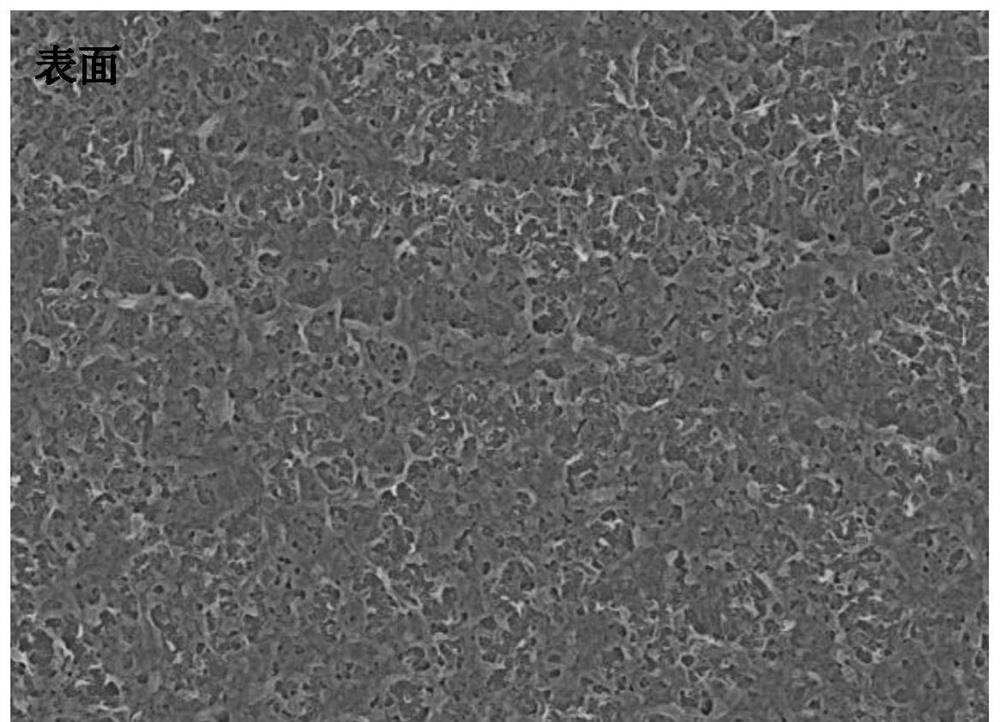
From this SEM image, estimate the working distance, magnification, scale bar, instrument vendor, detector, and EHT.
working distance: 6.5 mm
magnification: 10 K X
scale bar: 1000 nm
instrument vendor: Zeiss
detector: InLens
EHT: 2 kV